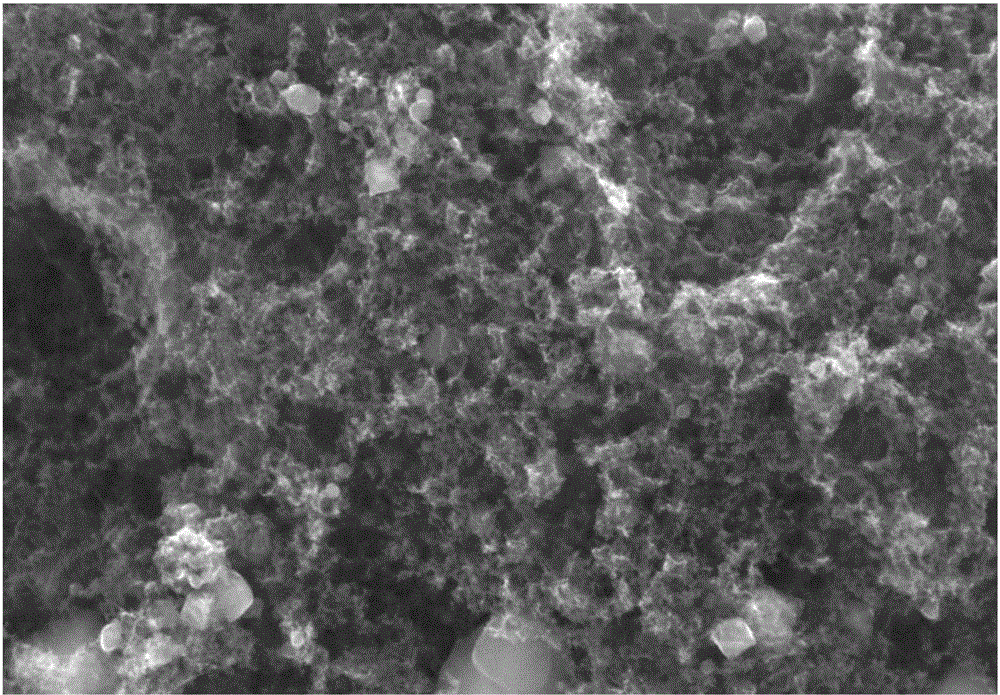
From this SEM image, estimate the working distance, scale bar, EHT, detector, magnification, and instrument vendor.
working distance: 9.8 mm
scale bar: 1000 nm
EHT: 20 kV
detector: ETD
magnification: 100 K X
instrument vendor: FEI